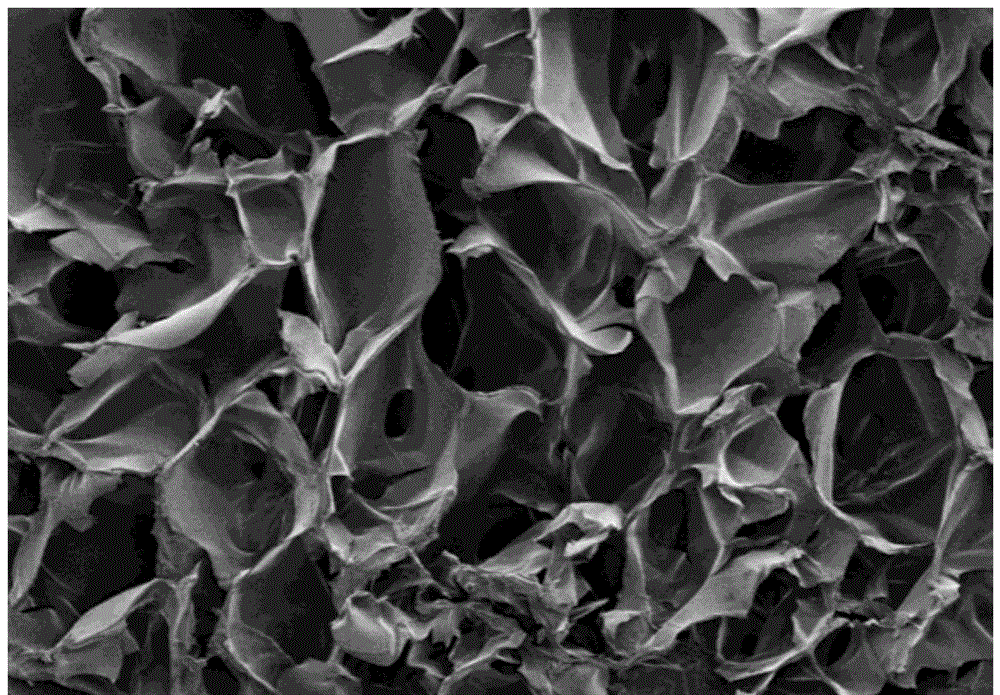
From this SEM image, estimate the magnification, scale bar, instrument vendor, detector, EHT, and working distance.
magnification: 0.1 K X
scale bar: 500000 nm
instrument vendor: Hitachi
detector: SE(M)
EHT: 3 kV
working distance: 8.9 mm